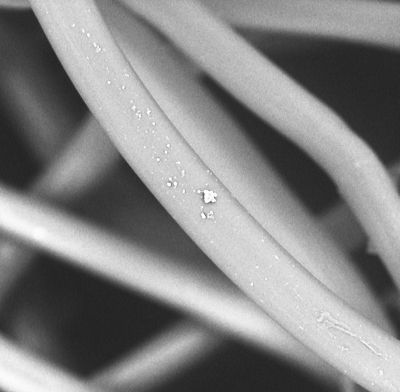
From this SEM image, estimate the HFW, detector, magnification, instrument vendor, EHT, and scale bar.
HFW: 136 µm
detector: BSD Full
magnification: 2 K X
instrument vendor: other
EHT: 10 kV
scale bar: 30000 nm